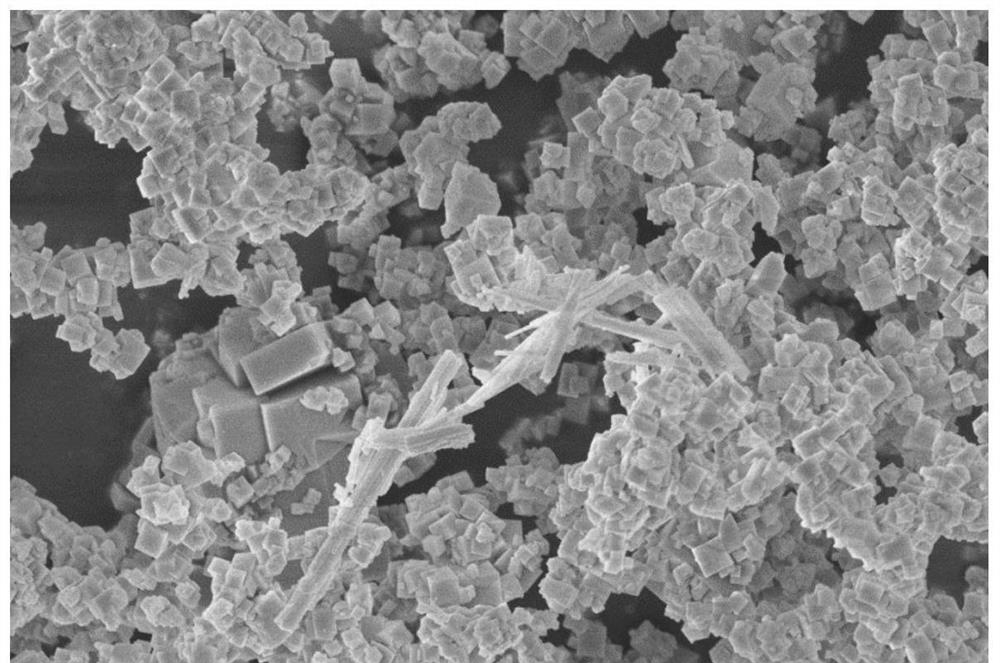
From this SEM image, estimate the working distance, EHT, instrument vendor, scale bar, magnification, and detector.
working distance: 6.1 mm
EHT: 5 kV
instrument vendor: Zeiss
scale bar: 1000 nm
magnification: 10 K X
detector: InLens